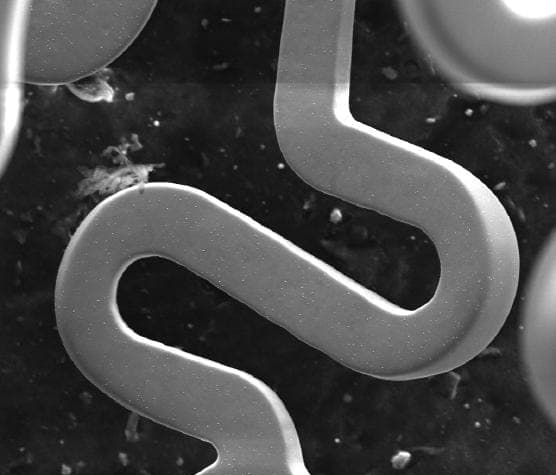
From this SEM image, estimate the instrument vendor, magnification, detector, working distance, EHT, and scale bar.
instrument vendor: FEI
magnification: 0.537 K X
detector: ETD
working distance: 11.5 mm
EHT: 25 kV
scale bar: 200000 nm